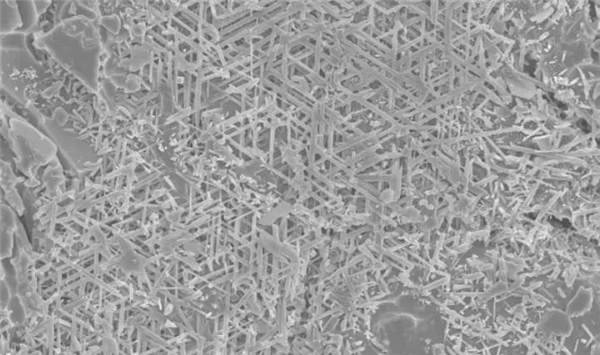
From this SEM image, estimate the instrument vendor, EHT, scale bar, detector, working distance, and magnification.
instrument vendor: Hitachi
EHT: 5 kV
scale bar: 10000 nm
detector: SE(UL)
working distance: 9.1 mm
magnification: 5 K X